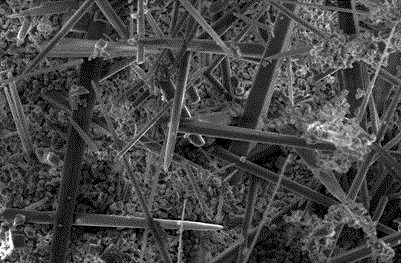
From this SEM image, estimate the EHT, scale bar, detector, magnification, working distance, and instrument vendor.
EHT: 15 kV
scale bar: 10000 nm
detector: SEI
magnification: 1 K X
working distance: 18 mm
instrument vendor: JEOL